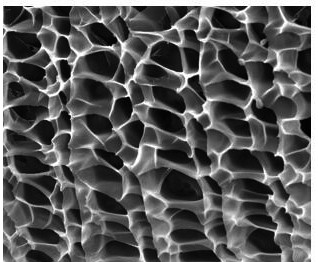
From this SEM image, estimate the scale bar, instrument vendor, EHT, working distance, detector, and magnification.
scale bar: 10000 nm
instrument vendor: FEI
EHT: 20 kV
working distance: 9.2 mm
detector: ETD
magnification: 10 K X